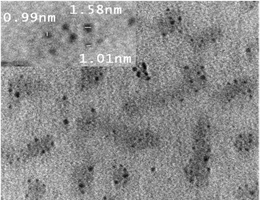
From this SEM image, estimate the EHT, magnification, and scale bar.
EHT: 200 kV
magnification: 100 K X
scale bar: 50 nm